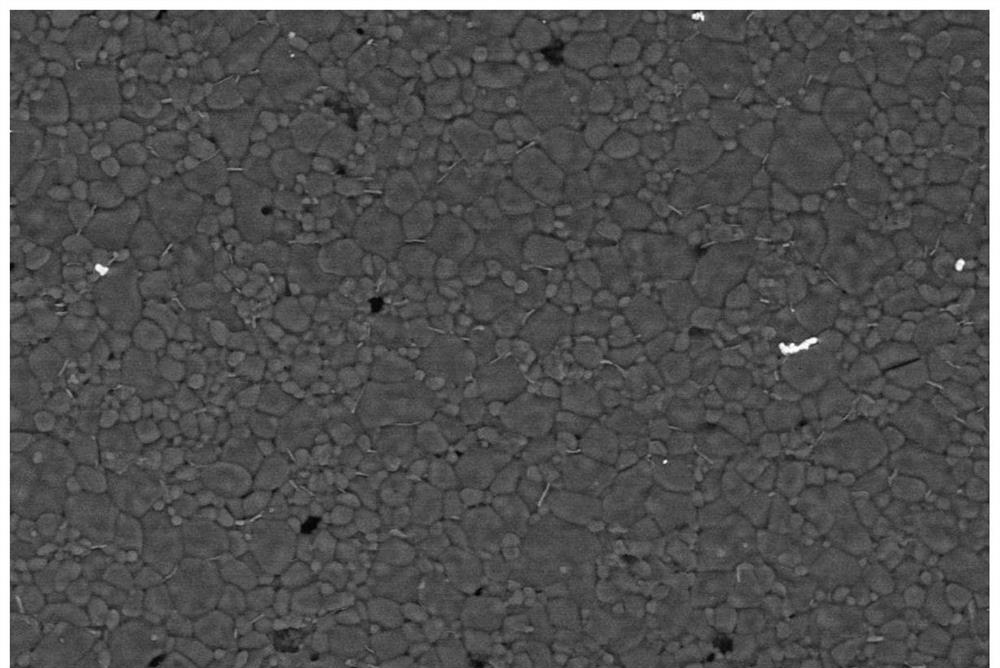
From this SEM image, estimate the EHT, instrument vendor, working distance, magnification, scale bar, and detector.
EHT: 20 kV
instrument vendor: Zeiss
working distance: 10 mm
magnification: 0.5 K X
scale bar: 10000 nm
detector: NTS BSD